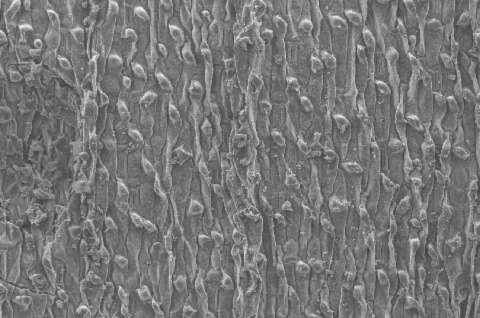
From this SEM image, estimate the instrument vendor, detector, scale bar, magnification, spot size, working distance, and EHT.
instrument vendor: FEI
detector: SE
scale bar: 100000 nm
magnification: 0.2 K X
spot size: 3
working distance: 10 mm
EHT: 10 kV